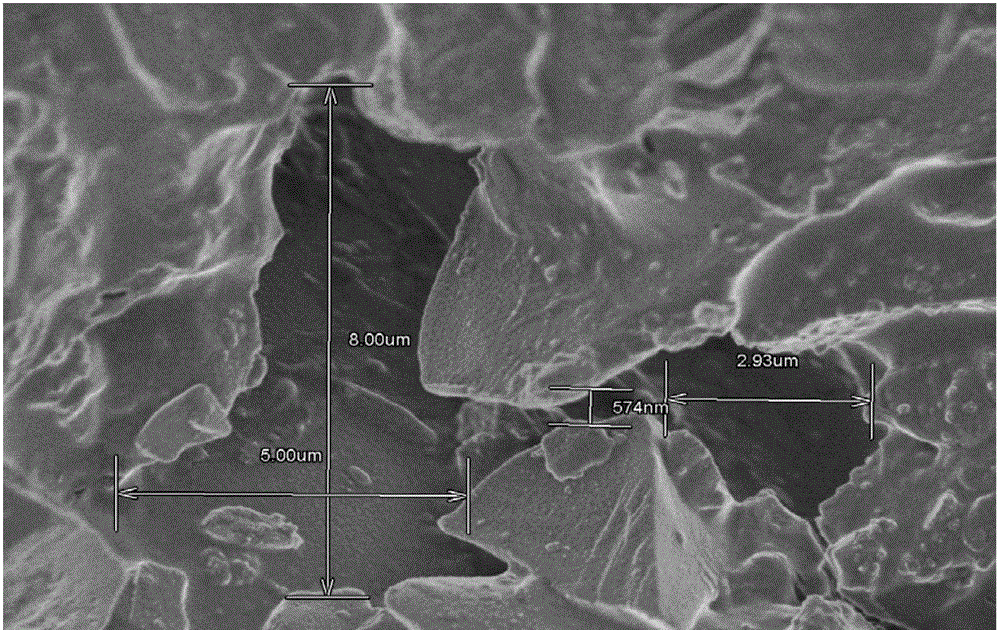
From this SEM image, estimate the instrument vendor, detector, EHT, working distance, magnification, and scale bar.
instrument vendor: Hitachi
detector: SE(M)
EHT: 2 kV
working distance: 4.3 mm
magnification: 9 K X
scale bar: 5000 nm